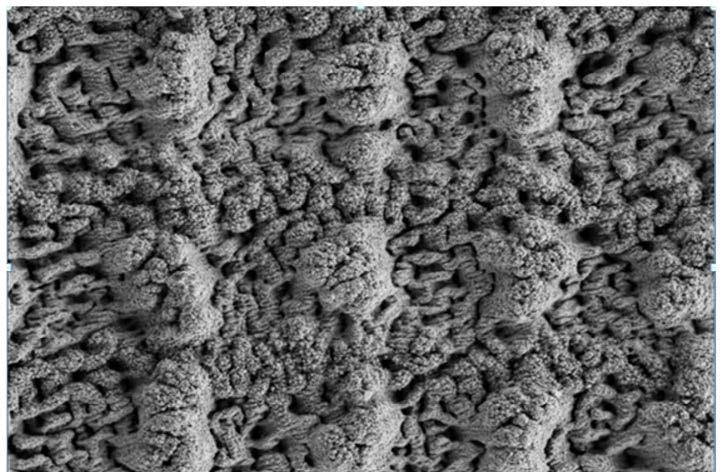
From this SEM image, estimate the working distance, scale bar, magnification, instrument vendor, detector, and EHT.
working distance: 5.1 mm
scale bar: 10000 nm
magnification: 1 K X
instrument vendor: Zeiss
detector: SE2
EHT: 3 kV